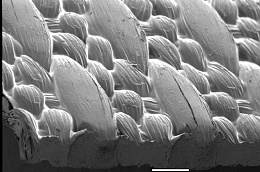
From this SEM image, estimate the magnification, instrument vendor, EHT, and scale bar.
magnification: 0.035 K X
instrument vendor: JEOL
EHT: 5 kV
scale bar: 500000 nm